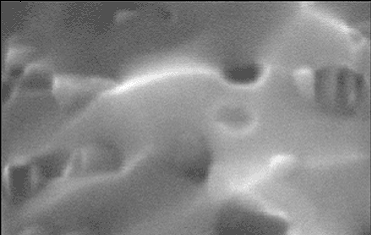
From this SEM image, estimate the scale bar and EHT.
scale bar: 500 nm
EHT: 20 kV